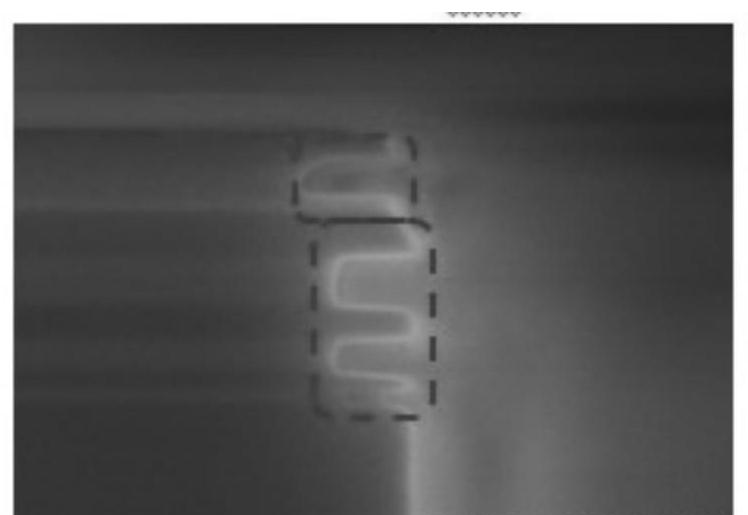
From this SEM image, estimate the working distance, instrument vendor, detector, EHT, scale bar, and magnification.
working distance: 0.6 mm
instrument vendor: Hitachi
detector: SE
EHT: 3 kV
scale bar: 200 nm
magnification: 250 K X